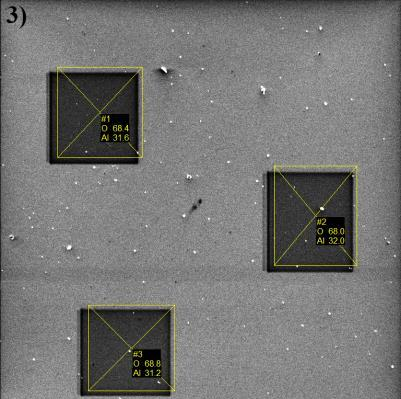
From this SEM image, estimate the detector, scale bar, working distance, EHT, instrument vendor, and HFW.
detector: SE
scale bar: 50000 nm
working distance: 15.27 mm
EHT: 5 kV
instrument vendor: Tescan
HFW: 218 µm